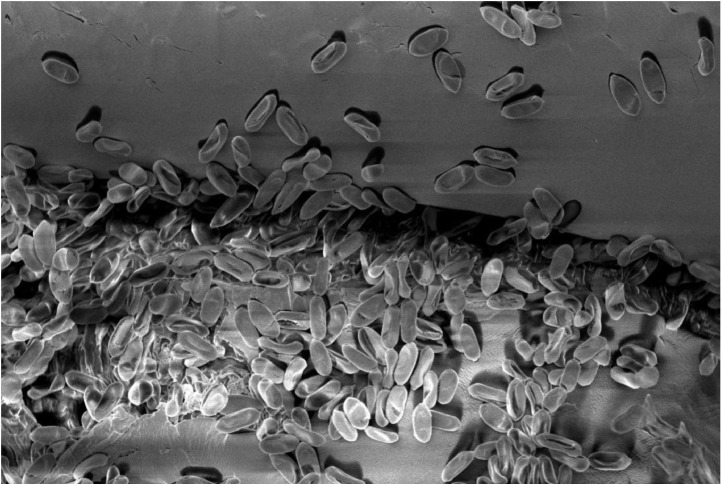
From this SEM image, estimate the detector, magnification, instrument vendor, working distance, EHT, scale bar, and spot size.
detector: SEI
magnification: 1.3 K X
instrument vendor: JEOL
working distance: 18 mm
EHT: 5 kV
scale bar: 10000 nm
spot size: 30